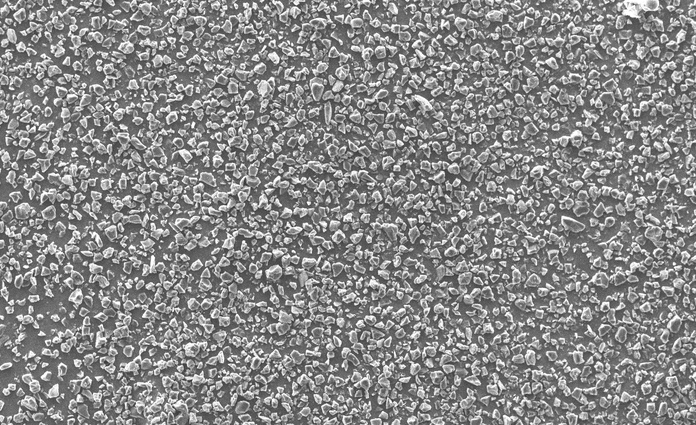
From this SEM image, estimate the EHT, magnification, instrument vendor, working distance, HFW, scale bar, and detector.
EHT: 10 kV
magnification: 1 K X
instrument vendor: Thermo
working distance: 5.738 mm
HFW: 519 µm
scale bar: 150000 nm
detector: SED